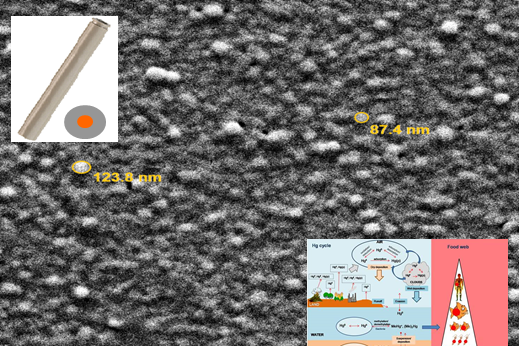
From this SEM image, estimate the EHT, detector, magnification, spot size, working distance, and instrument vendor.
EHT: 10 kV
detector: ETD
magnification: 80 K X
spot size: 3.5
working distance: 11.7 mm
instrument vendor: FEI